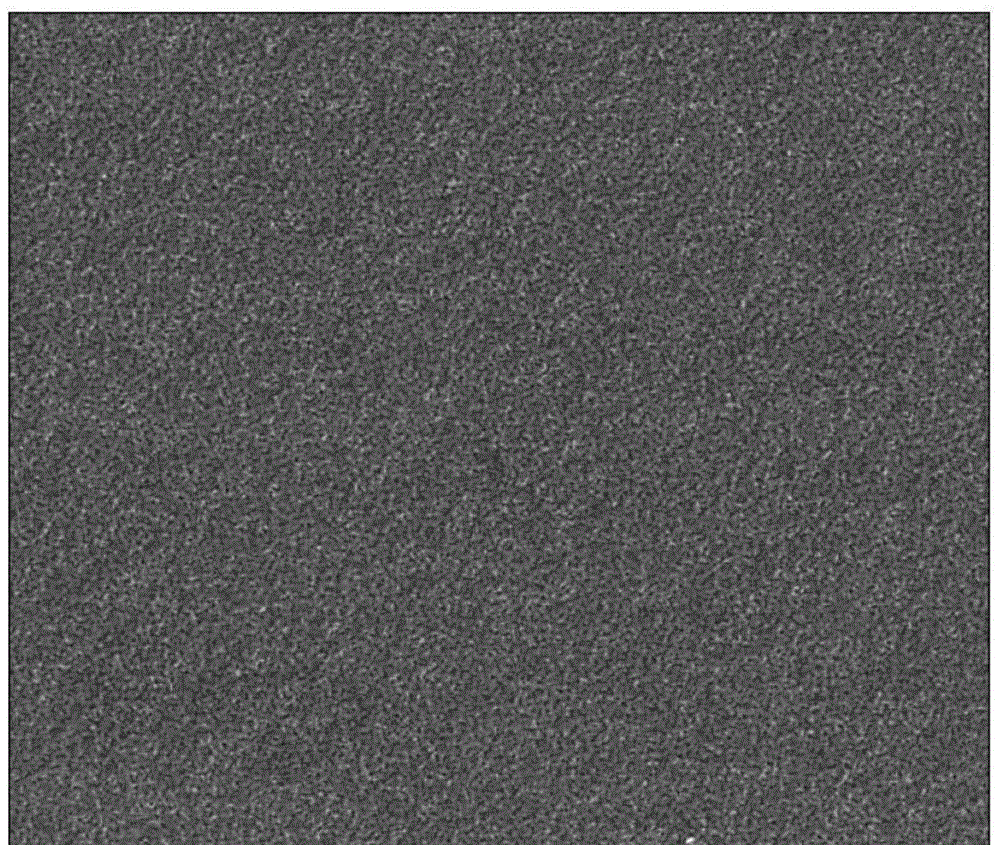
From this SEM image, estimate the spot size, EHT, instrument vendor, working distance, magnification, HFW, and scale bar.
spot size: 4.5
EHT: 5 kV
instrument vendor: FEI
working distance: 10 mm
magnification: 10 K X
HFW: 14.9 µm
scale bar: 5000 nm